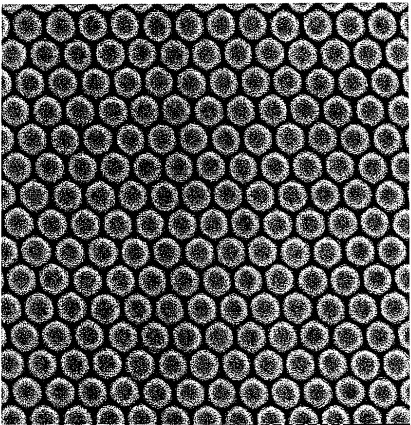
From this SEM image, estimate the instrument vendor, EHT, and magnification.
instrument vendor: JEOL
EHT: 5 kV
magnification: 30 K X